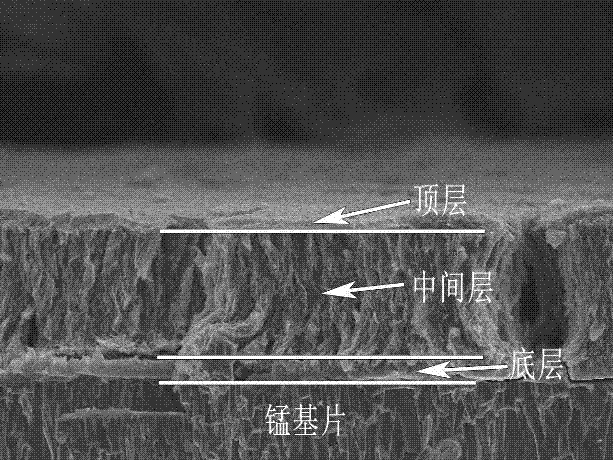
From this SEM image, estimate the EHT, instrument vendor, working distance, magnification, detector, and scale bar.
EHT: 10 kV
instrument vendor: JEOL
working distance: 12.2 mm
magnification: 2.5 K X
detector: SEI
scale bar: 10000 nm